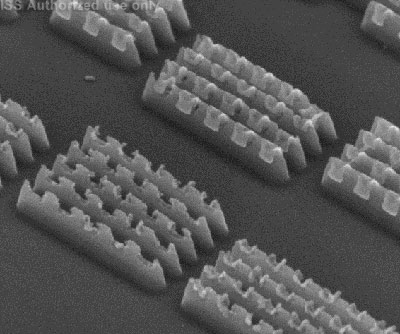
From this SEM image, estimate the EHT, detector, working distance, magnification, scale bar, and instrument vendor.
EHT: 5 kV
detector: TLD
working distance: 4.1 mm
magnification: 250 K X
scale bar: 400 nm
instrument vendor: FEI